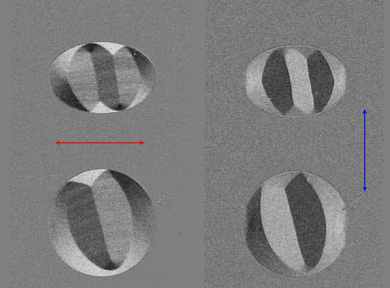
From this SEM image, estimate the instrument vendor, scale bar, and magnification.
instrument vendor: other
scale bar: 100000 nm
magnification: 0.02 K X